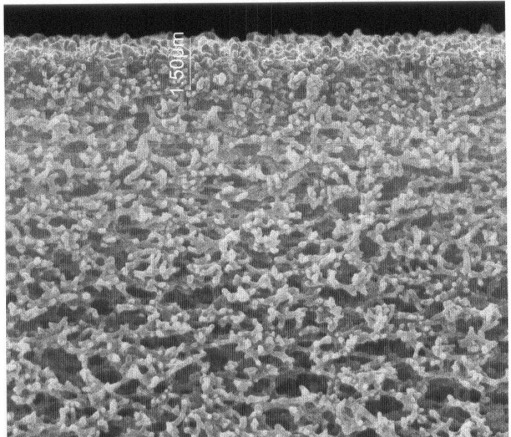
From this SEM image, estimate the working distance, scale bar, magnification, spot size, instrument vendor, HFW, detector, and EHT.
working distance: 9.3 mm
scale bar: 5000 nm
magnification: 20 K X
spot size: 2.5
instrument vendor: FEI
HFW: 14.9 µm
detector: ETD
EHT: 30 kV